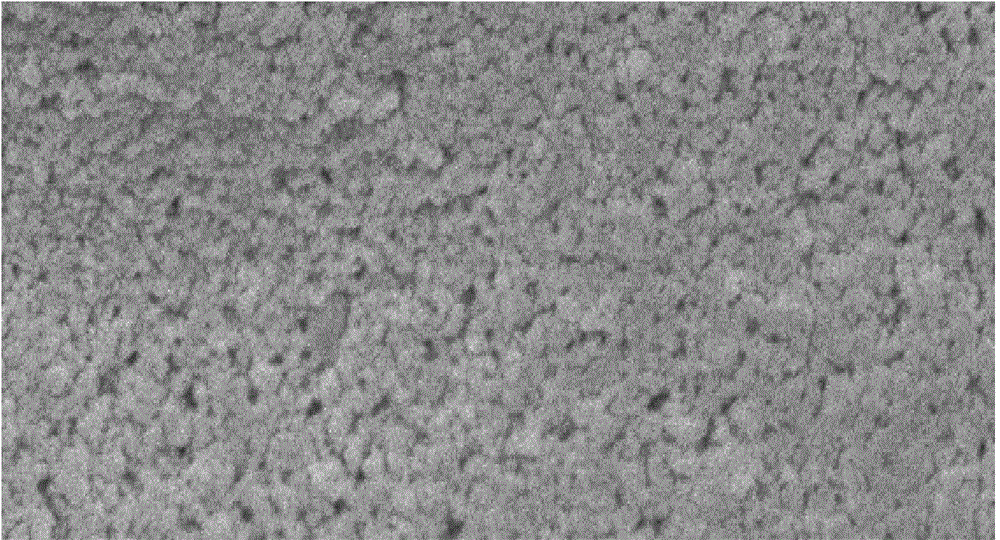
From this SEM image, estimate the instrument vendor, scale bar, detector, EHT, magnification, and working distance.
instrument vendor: JEOL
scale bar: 1000 nm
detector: SEI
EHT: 5 kV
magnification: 10 K X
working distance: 9.4 mm